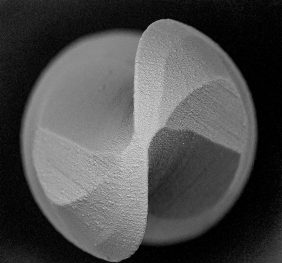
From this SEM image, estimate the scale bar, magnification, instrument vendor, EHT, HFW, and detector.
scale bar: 100000 nm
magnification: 0.47 K X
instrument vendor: other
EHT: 15 kV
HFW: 374 µm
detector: BSD full